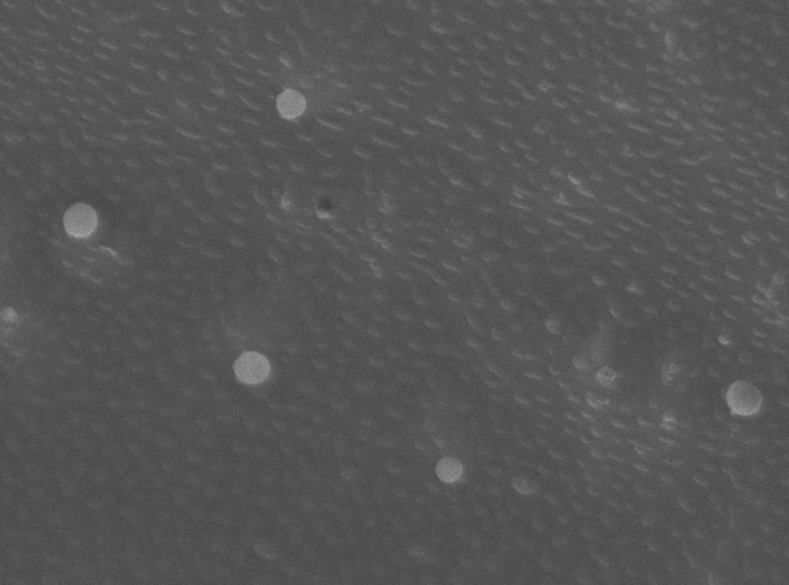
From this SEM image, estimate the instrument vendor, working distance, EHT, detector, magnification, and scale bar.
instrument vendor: JEOL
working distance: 5.7 mm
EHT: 5 kV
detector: SEI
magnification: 33 K X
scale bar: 100 nm